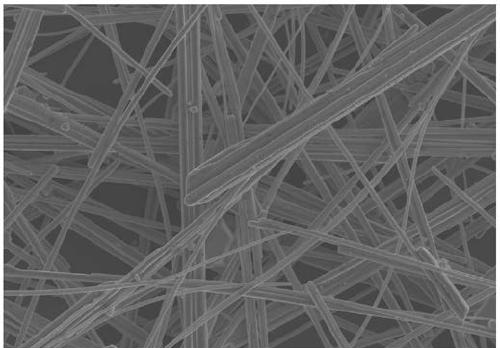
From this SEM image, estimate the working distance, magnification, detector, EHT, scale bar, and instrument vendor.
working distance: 3.8 mm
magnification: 10 K X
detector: InLens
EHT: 5 kV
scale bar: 1000 nm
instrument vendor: Zeiss